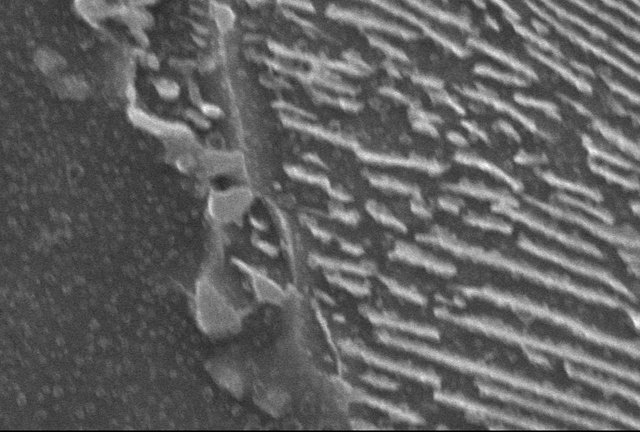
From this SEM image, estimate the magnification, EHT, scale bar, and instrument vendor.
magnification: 10 K X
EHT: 25 kV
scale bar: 1000 nm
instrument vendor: JEOL